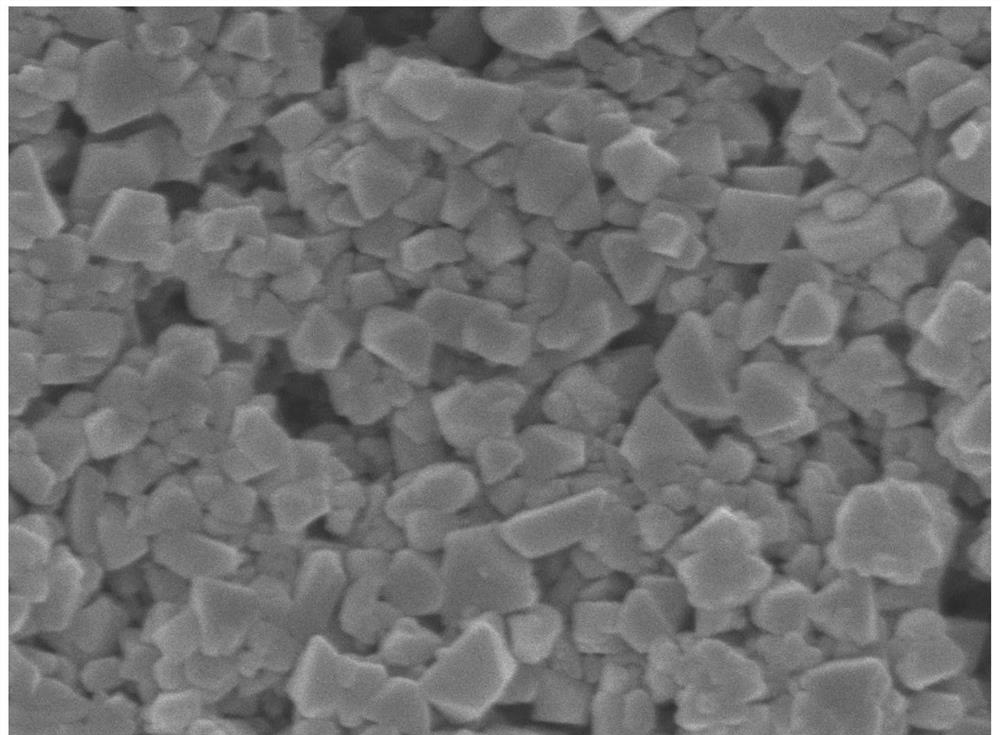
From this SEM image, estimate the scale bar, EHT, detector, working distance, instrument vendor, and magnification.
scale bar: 100 nm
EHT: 5 kV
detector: SEI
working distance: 8.2 mm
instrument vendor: JEOL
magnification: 30 K X